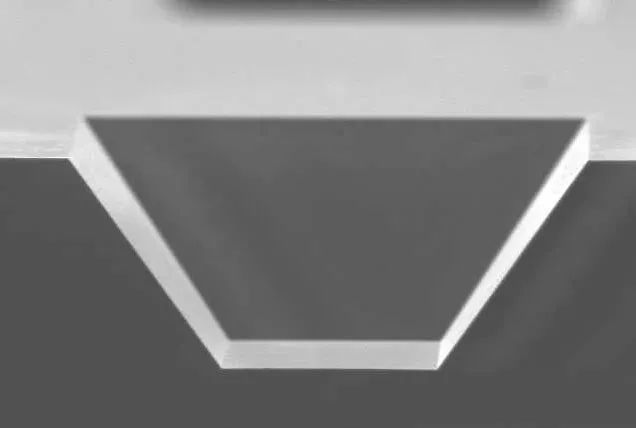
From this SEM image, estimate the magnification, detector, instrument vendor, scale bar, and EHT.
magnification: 0.13 K X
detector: SEI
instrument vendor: JEOL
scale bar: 100000 nm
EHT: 25 kV